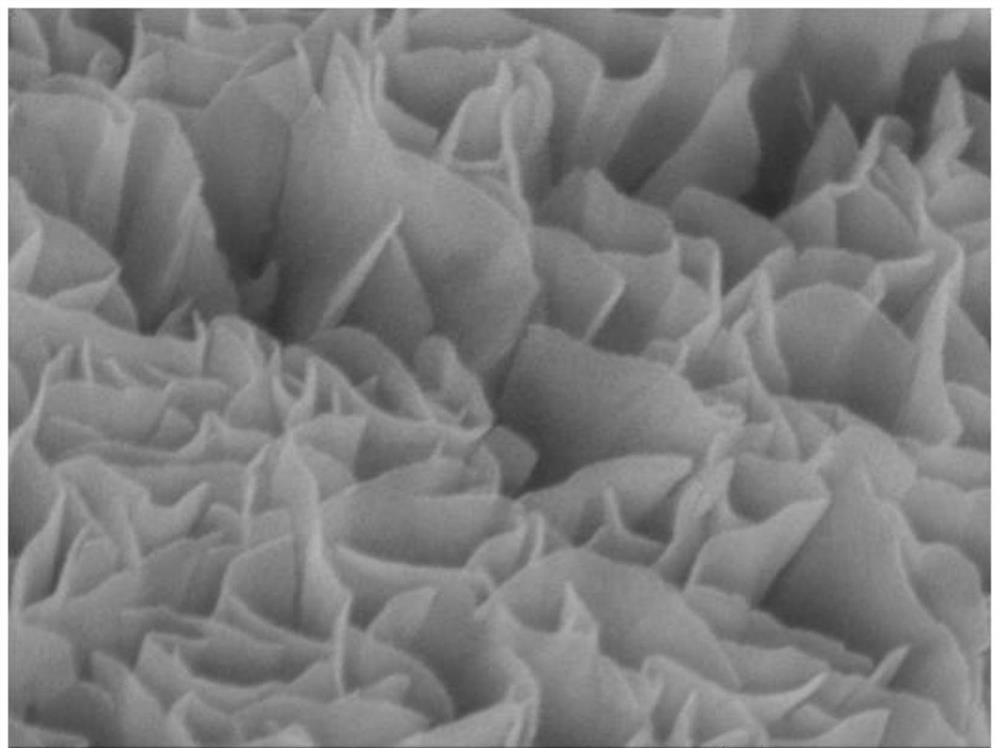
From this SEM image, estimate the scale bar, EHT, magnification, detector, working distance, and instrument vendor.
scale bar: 100 nm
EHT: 8 kV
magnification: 60 K X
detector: SEI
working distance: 8.9 mm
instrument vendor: JEOL